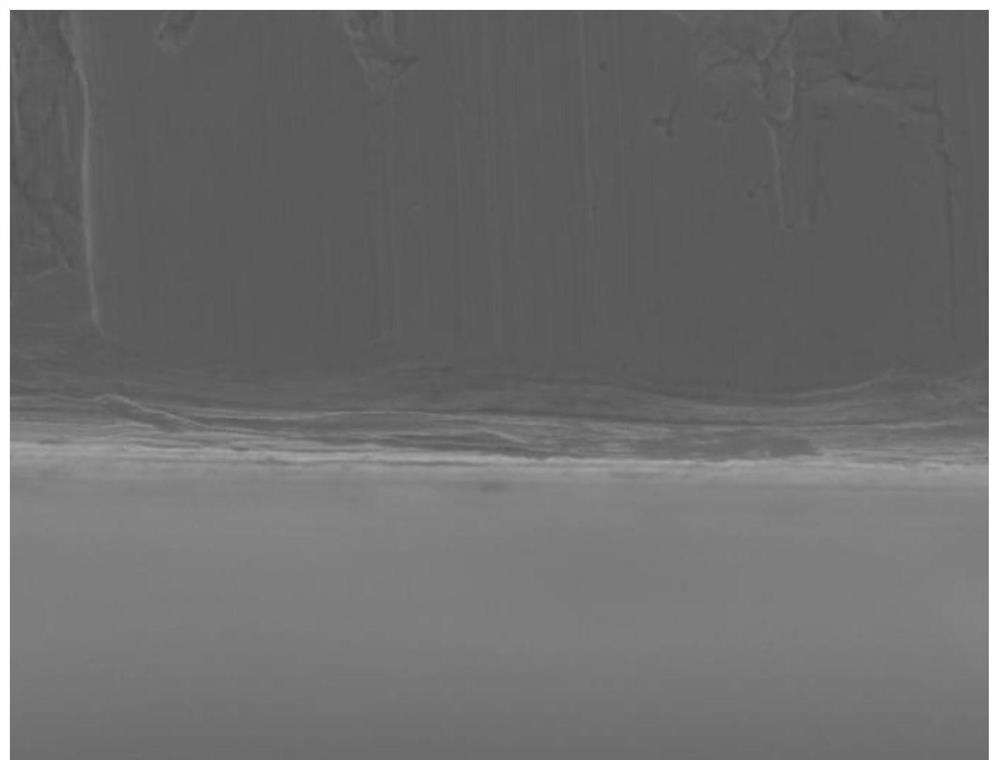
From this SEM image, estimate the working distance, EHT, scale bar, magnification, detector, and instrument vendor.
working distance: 11.1 mm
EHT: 20 kV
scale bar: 10000 nm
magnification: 1 K X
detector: LED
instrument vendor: JEOL